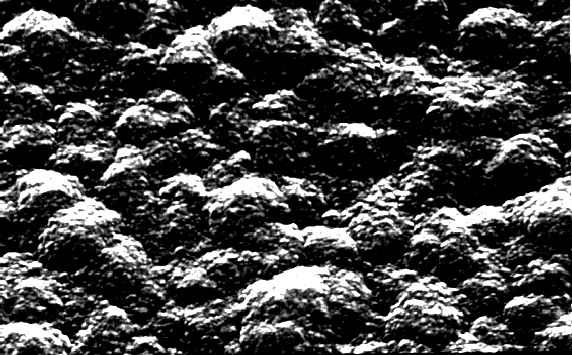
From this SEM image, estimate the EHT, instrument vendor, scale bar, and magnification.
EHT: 25 kV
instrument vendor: JEOL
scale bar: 5000 nm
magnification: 10 K X